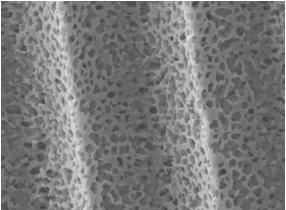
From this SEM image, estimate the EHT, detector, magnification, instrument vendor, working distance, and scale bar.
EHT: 10 kV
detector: LEI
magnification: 2.5 K X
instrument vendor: JEOL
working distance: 8 mm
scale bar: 10000 nm